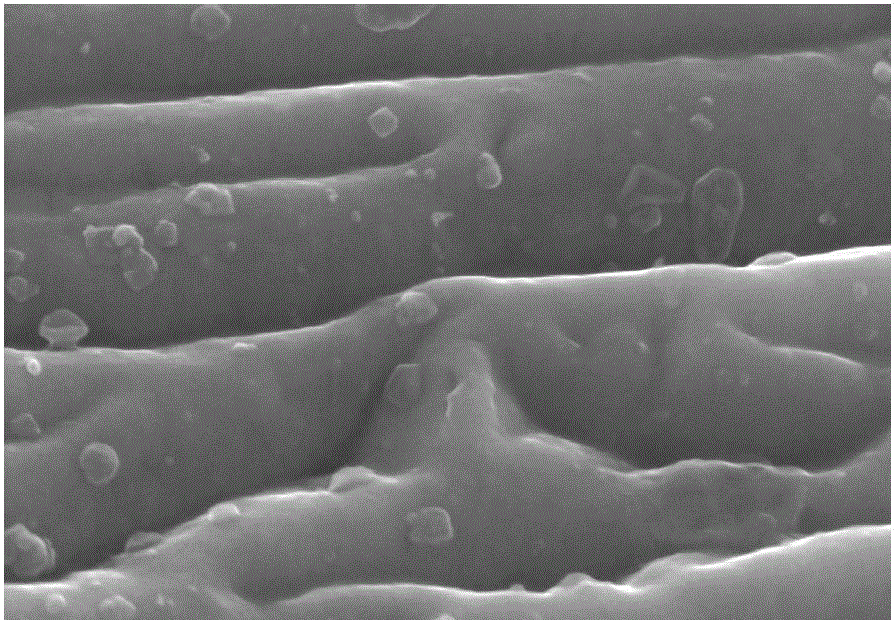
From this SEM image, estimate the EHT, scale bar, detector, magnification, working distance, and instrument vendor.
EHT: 15 kV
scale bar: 500 nm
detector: SE(U)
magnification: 80 K X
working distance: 9 mm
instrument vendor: Hitachi